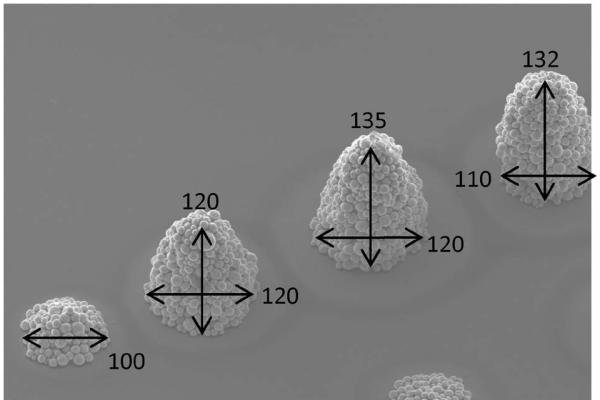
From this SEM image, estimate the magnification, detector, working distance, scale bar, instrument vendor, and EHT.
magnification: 0.2 K X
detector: SE(U)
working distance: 12 mm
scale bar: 200000 nm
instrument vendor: Hitachi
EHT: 15 kV